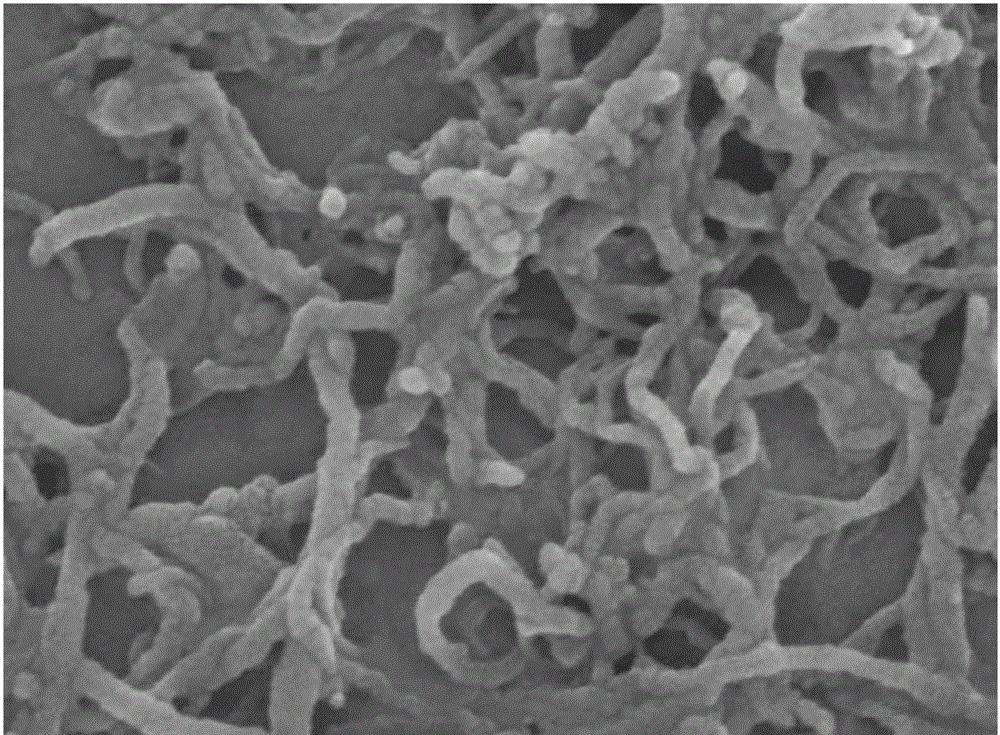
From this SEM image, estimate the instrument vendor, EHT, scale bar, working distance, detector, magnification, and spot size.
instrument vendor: FEI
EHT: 20 kV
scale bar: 500 nm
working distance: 5.2 mm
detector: TLD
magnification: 40 K X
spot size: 5